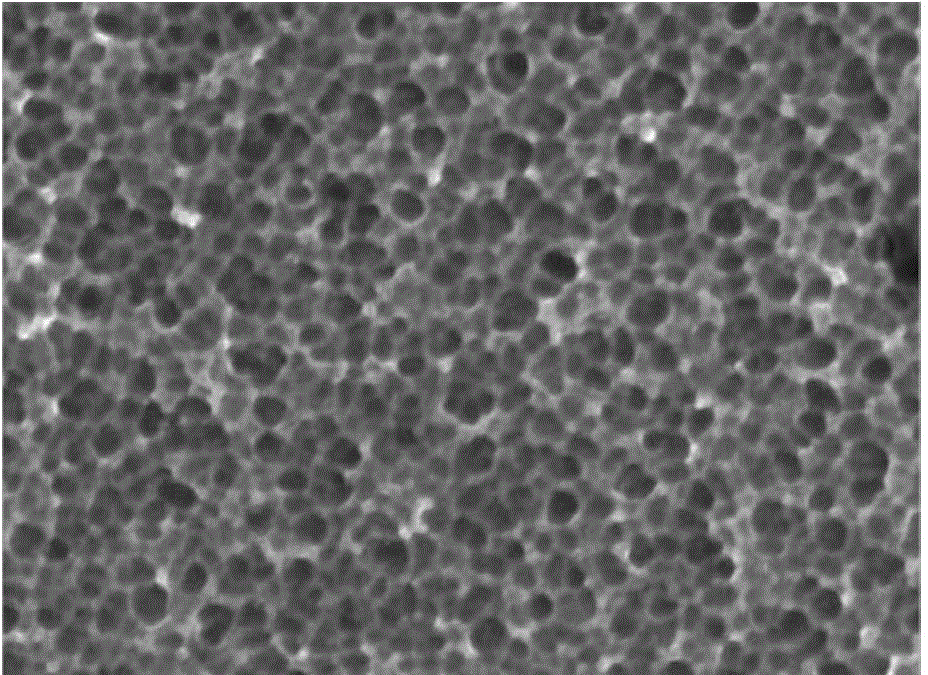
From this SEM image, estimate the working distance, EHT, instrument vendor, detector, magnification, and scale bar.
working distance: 6.8 mm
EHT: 10 kV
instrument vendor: JEOL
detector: SEI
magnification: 30 K X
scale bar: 100 nm